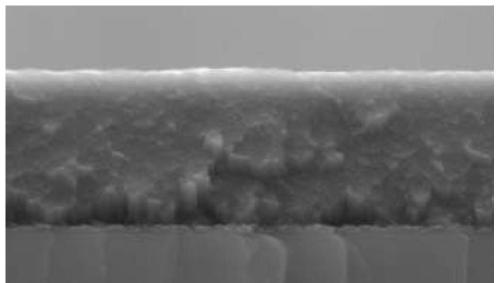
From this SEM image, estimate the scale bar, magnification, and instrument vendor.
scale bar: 2000 nm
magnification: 40 K X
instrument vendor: FEI